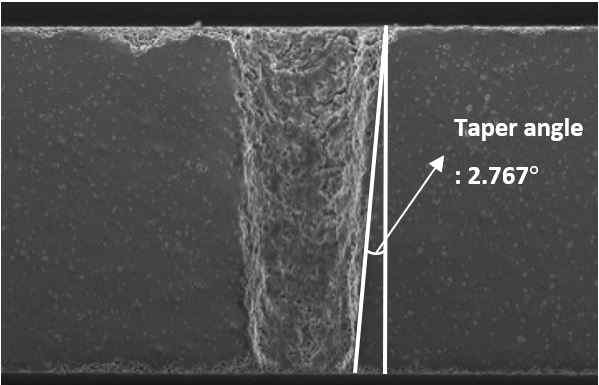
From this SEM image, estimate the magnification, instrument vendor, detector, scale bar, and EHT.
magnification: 0.25 K X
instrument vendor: JEOL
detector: SEI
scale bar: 100000 nm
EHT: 20 kV